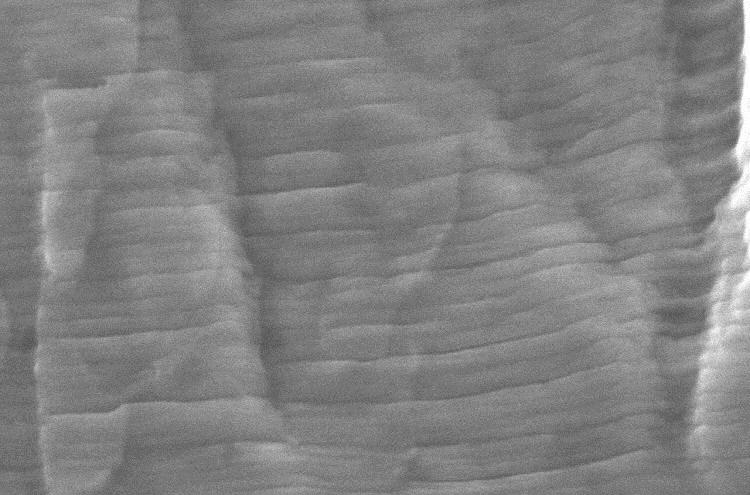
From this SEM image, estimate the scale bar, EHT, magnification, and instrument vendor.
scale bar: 1000 nm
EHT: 15 kV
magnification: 10 K X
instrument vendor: JEOL